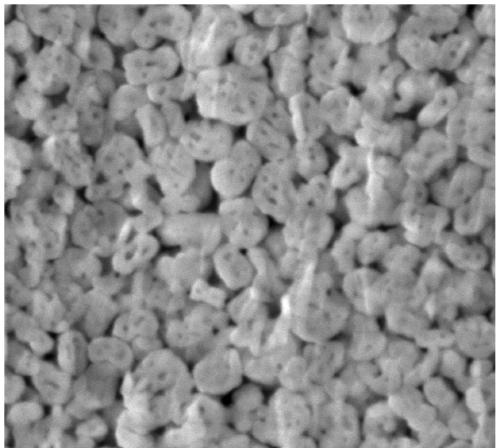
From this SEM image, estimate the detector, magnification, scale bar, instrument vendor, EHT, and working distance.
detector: SEI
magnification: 60 K X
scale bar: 100 nm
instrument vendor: JEOL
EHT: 5 kV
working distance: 12 mm